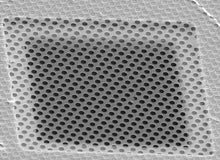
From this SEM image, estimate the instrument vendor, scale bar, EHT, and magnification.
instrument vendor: other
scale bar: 20000 nm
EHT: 25 kV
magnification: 1.5 K X